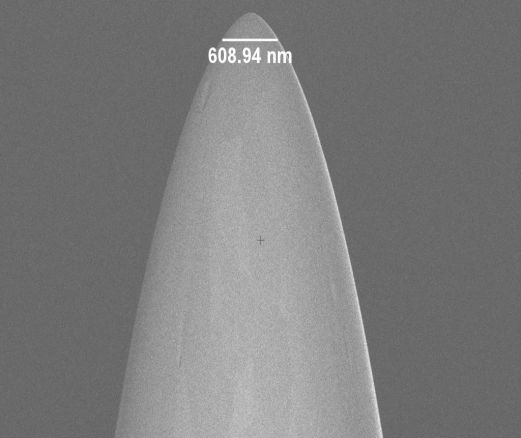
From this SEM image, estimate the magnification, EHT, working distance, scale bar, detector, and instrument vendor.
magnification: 20 K X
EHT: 2 kV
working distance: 4.6 mm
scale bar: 2000 nm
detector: InLens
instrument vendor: Zeiss